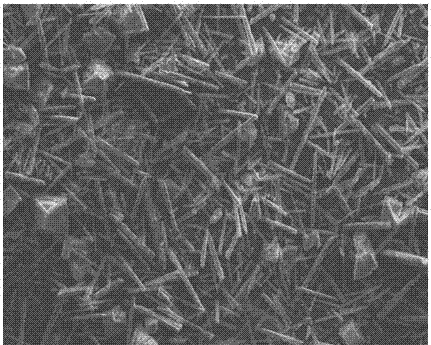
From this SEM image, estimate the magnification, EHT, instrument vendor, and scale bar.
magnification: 0.2 K X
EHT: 15 kV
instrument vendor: FEI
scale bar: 50000 nm